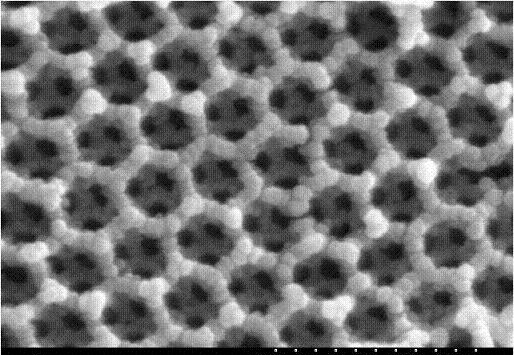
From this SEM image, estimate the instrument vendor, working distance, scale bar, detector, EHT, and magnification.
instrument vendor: Hitachi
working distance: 15.2 mm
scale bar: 500 nm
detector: SE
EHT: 20 kV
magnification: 100 K X